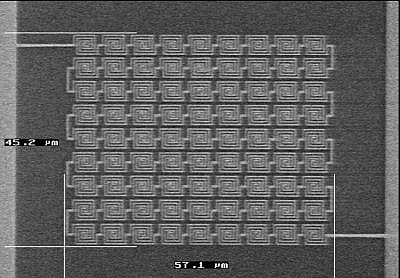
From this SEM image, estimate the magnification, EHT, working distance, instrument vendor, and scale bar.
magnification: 1.45 K X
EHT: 15 kV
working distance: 18 mm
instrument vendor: JEOL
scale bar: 20000 nm